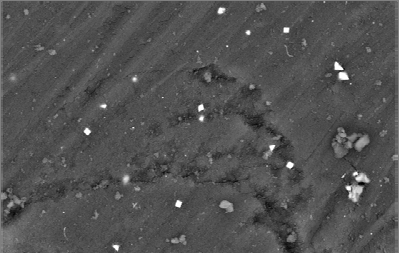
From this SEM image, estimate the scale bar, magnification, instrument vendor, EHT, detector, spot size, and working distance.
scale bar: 20000 nm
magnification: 1 K X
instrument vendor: FEI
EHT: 25 kV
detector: BSE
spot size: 4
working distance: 10.5 mm